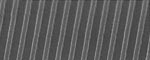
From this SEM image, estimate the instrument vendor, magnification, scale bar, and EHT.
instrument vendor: Hitachi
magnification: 50 K X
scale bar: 600 nm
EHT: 5 kV